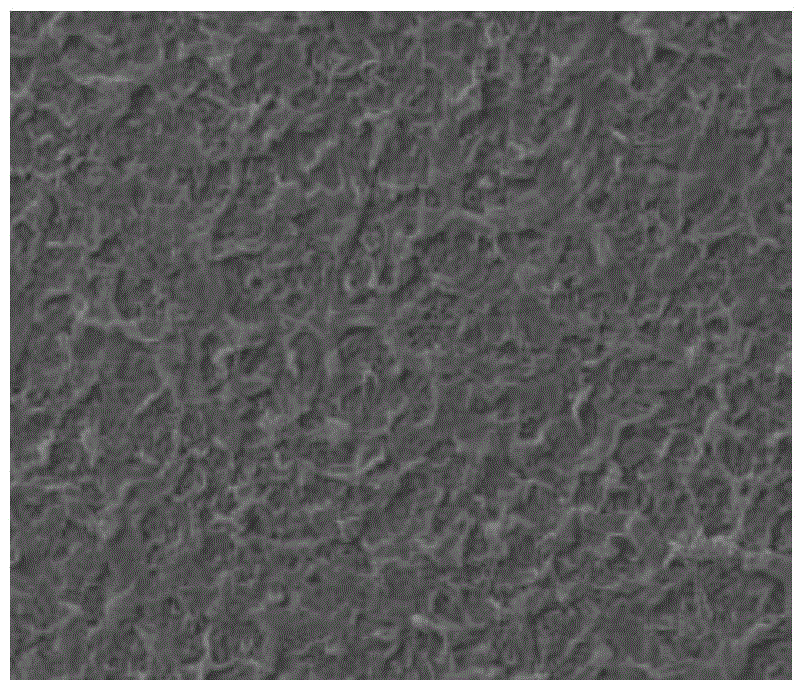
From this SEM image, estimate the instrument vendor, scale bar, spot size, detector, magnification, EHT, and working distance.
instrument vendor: FEI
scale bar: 2000 nm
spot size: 2.5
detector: TLD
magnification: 30 K X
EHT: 10 kV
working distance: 5.5 mm